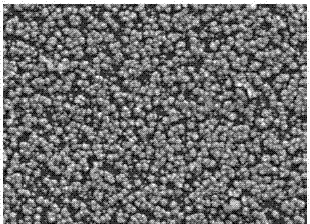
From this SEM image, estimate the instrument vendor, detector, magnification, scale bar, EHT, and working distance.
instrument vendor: JEOL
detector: LED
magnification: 20 K X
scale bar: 1000 nm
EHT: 5 kV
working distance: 3.7 mm